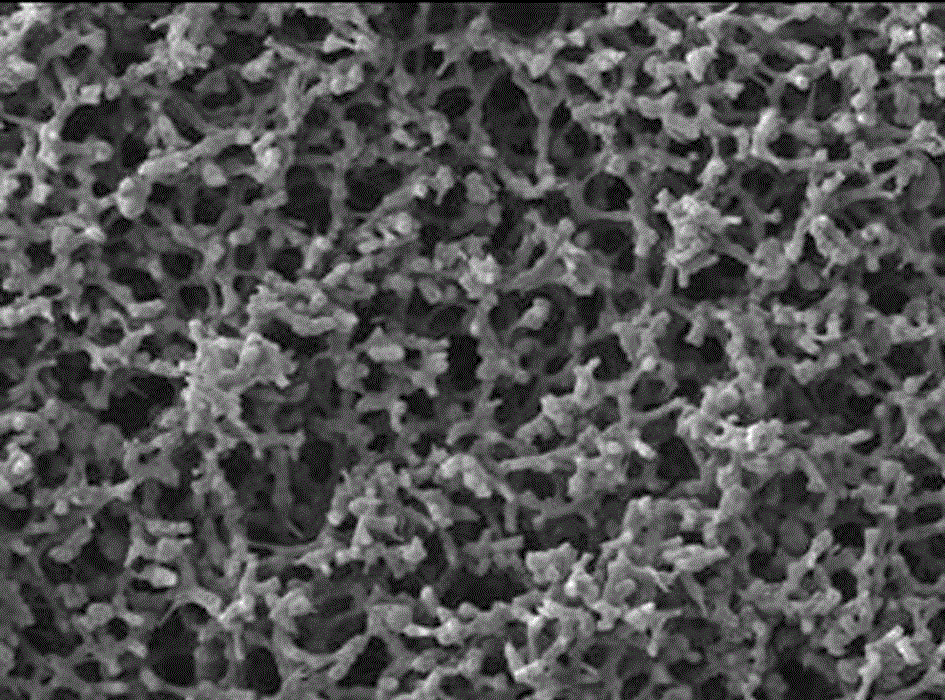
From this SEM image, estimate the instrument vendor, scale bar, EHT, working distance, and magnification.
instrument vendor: Hitachi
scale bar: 10000 nm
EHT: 15 kV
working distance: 8 mm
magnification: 0.5 K X